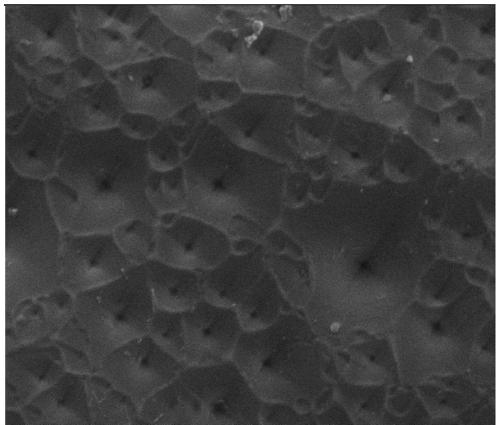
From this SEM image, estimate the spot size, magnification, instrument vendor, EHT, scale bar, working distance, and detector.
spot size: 3.5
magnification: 10 K X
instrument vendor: FEI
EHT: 15 kV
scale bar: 5000 nm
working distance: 7.1 mm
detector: ETD